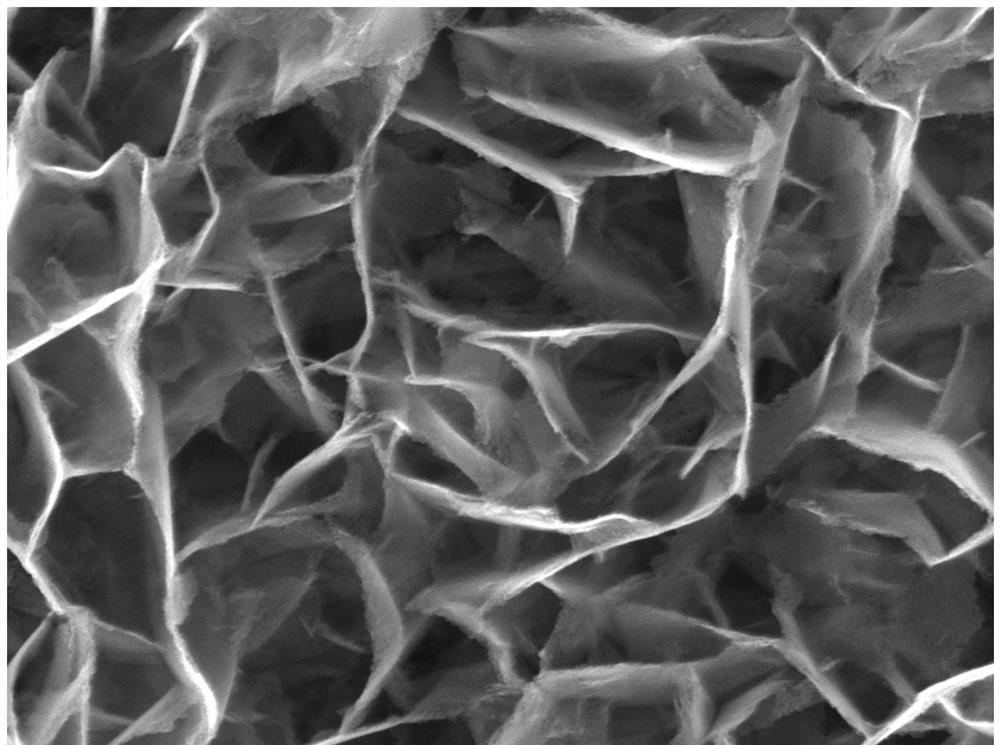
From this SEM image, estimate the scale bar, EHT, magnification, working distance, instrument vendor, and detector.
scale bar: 1000 nm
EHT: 20 kV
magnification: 50 K X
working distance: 15.96 mm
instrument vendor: Tescan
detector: SE